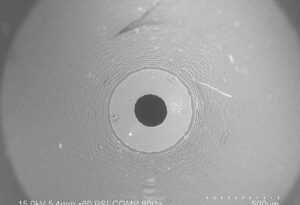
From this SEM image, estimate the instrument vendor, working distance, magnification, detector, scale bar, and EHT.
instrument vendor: Hitachi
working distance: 5.4 mm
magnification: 0.06 K X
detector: BSECOMP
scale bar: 500000 nm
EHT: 15 kV